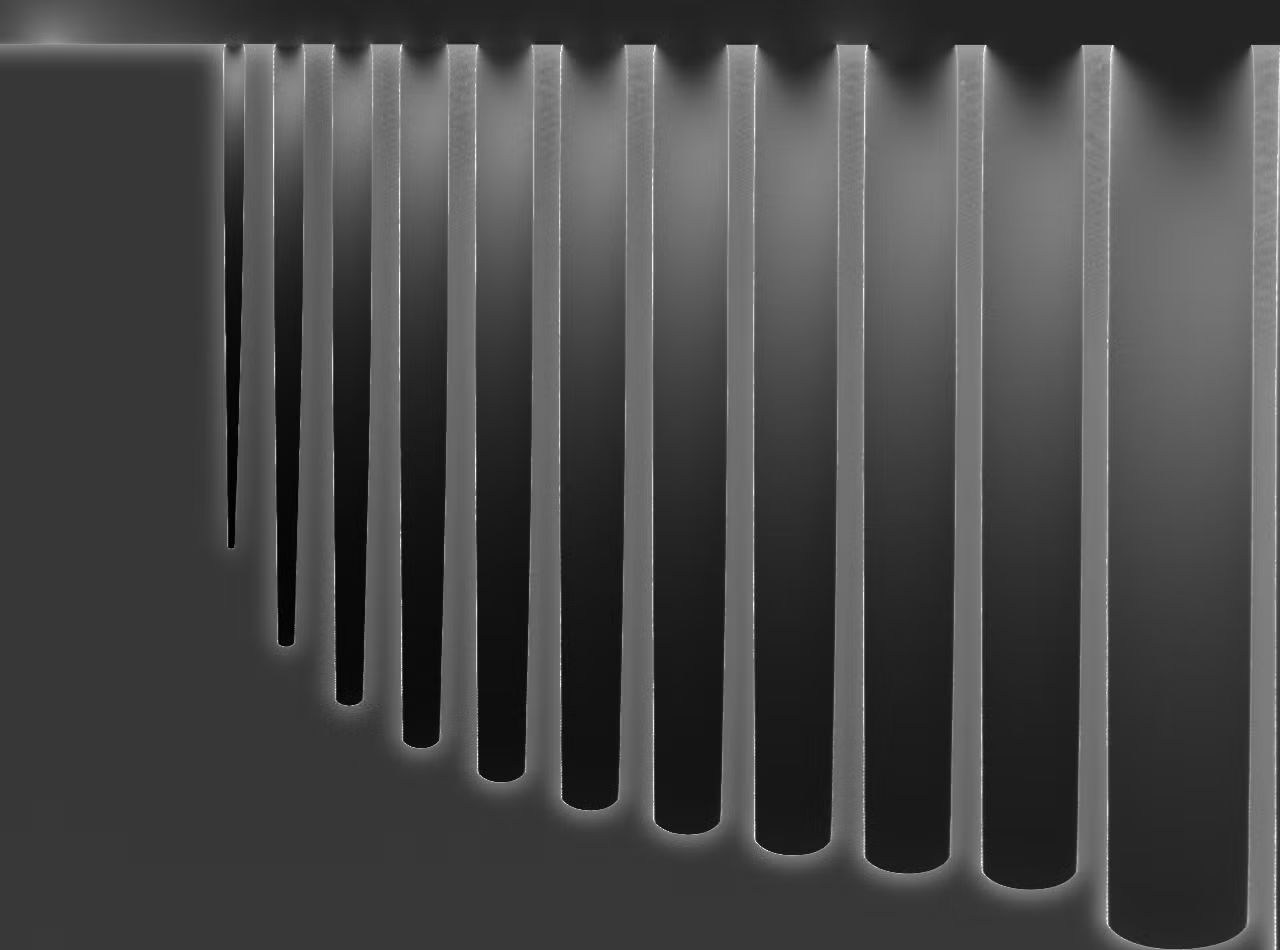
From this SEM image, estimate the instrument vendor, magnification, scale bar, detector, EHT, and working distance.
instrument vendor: JEOL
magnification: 0.8 K X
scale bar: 10000 nm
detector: LED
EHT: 20 kV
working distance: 5.5 mm